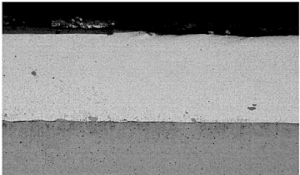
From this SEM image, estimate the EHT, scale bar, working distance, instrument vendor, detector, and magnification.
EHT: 20 kV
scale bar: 200000 nm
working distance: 23 mm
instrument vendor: Zeiss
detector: QBSD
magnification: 0.05 K X